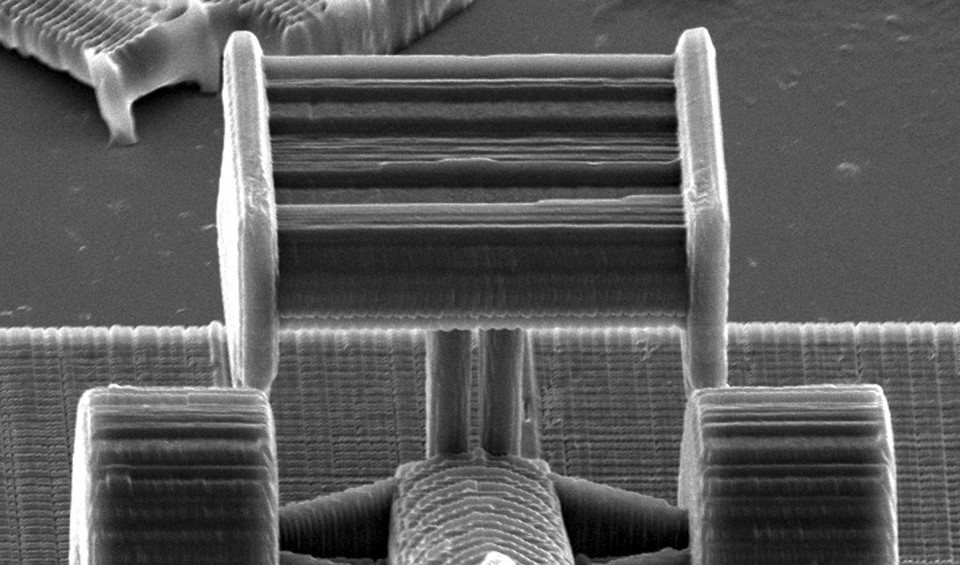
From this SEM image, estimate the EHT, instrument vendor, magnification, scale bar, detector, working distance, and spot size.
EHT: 20 kV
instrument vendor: FEI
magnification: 0.836 K X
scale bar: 20000 nm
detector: SE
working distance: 27.2 mm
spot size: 4